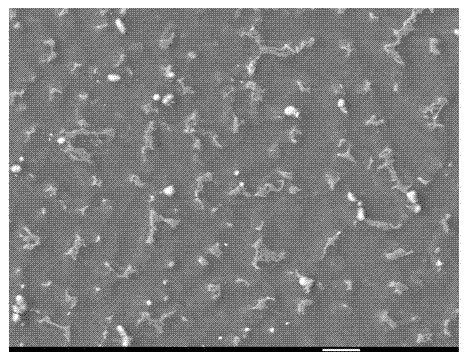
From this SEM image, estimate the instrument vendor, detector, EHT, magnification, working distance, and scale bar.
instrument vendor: JEOL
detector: LEI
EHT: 15 kV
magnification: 1 K X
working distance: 15.3 mm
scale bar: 10000 nm